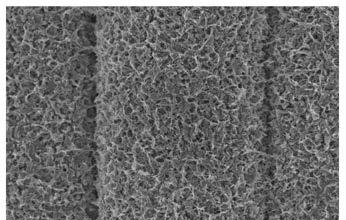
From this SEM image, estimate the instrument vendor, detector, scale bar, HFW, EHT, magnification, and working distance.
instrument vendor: FEI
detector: TLD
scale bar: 4000 nm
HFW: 20.7 µm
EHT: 2 kV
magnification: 20 K X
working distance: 3.6 mm